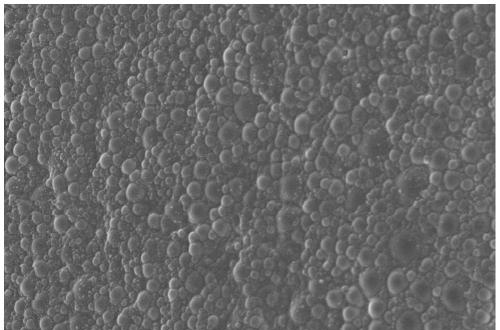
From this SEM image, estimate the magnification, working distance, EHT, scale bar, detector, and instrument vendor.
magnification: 9.49 K X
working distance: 7.4 mm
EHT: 5 kV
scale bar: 2000 nm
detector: InLens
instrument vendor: Zeiss